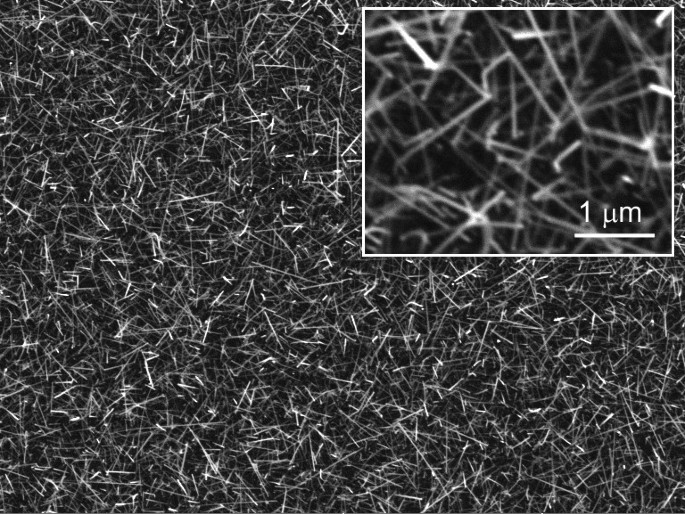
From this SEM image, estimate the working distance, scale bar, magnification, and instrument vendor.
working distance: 2.4471 mm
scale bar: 10000 nm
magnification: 6.58 K X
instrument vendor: Tescan